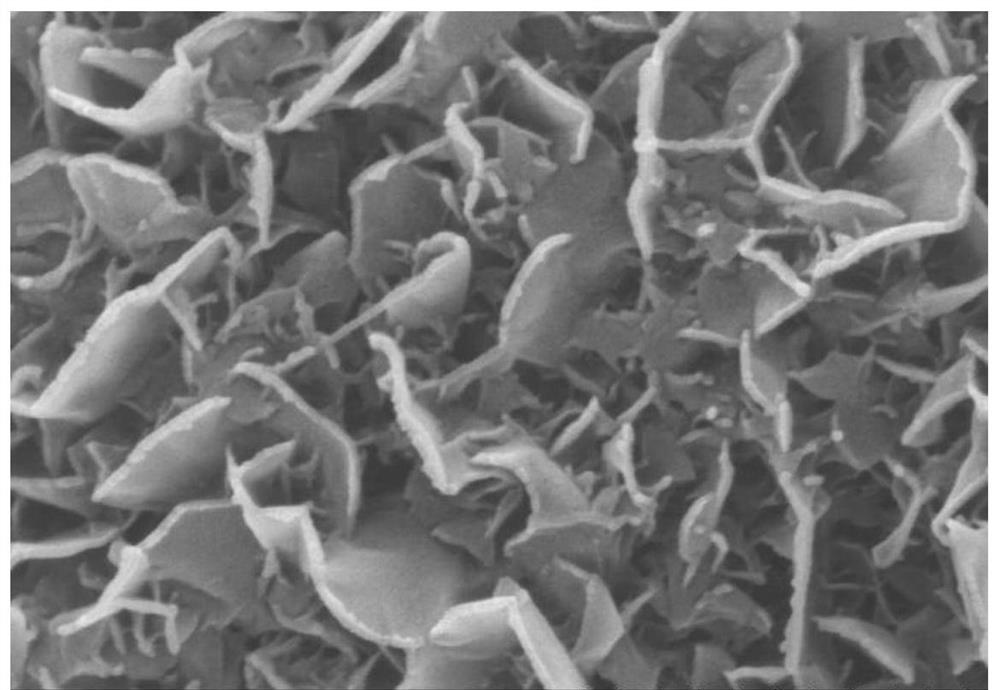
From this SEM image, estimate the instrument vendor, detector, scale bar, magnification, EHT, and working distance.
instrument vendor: Hitachi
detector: SE(M)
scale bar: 500 nm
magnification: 100 K X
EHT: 5 kV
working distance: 7 mm